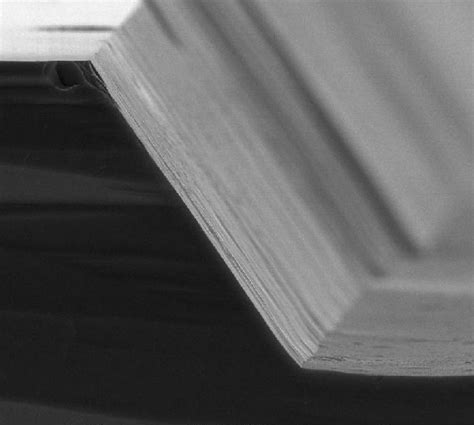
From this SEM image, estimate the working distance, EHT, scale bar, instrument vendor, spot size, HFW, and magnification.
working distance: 11.4 mm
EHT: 25 kV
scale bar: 50000 nm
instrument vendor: FEI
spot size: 3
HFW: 165 µm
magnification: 1.613 K X